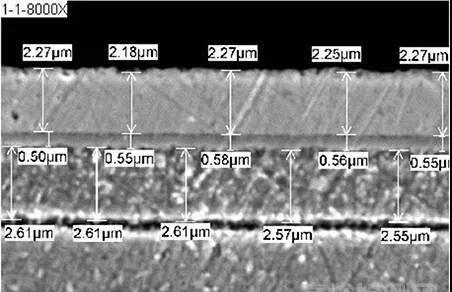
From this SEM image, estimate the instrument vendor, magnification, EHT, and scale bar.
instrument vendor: JEOL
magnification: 8 K X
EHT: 15 kV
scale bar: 2000 nm